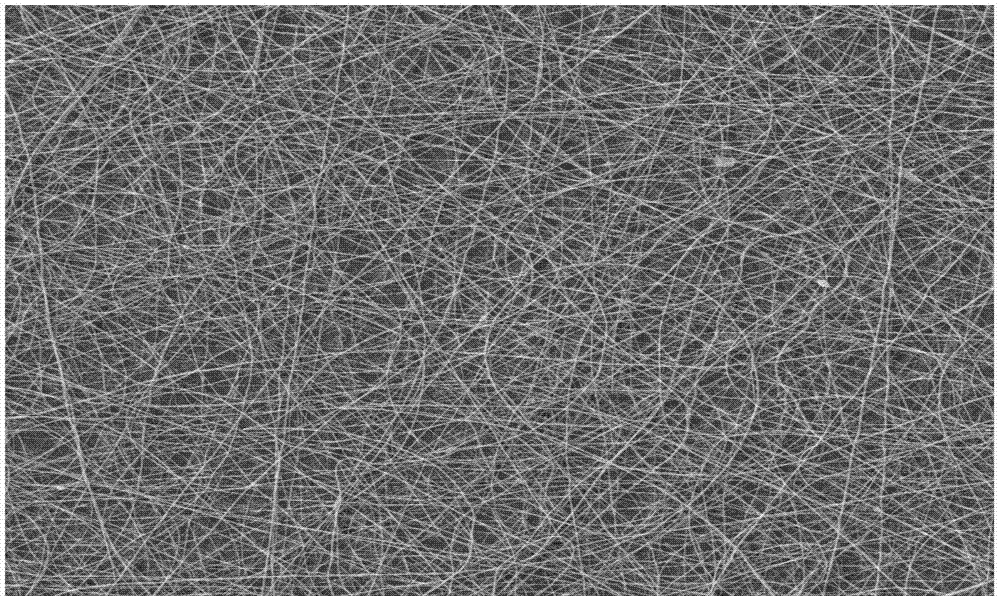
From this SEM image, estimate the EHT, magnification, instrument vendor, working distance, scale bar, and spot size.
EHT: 20 kV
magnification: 1 K X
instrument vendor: FEI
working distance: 7.3 mm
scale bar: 100000 nm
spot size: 3